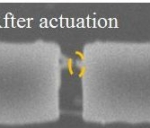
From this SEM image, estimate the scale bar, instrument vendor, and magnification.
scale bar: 500 nm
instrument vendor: JEOL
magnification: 50 K X